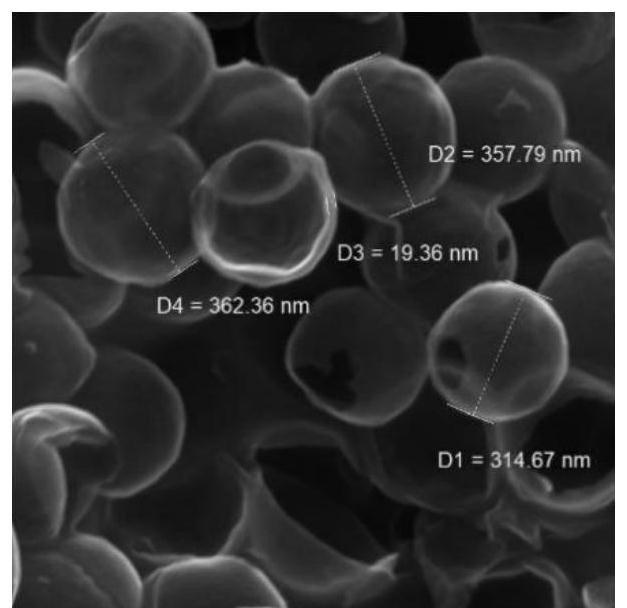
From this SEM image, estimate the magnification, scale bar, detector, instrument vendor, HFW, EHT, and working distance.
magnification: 150 K X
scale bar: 200 nm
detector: In-Beam SE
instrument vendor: Tescan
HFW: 1.38 µm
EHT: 10 kV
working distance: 5.19 mm